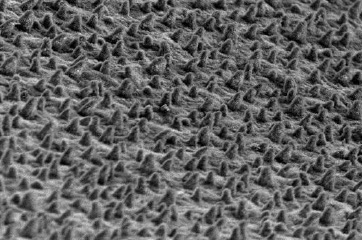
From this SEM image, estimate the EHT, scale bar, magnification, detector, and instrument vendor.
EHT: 10 kV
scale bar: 50000 nm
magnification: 0.5 K X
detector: SEI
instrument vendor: JEOL